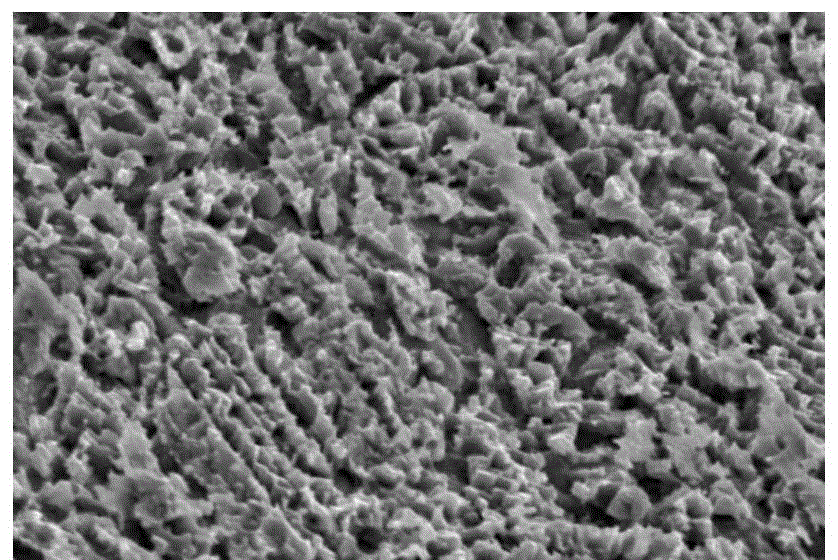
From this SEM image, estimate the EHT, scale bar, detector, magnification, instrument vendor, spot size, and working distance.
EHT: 20 kV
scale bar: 5000 nm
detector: SEI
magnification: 3.5 K X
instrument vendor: JEOL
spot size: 40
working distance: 25 mm